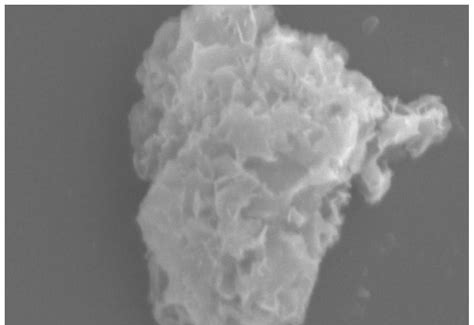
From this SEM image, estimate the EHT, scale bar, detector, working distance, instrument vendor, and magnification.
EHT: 15 kV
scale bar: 10000 nm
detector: SE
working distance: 40.4 mm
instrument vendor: Hitachi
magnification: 4 K X